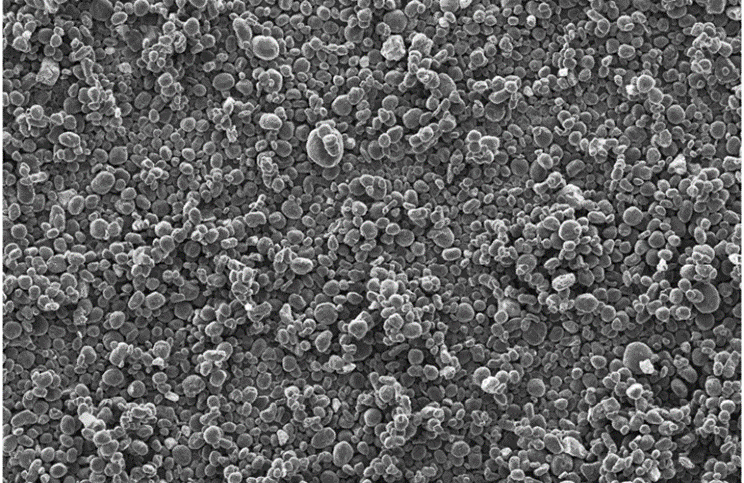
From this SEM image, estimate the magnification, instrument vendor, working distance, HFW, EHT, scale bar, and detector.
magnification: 0.5 K X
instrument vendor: FEI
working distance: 10 mm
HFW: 829 µm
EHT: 10 kV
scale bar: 200000 nm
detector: ETD